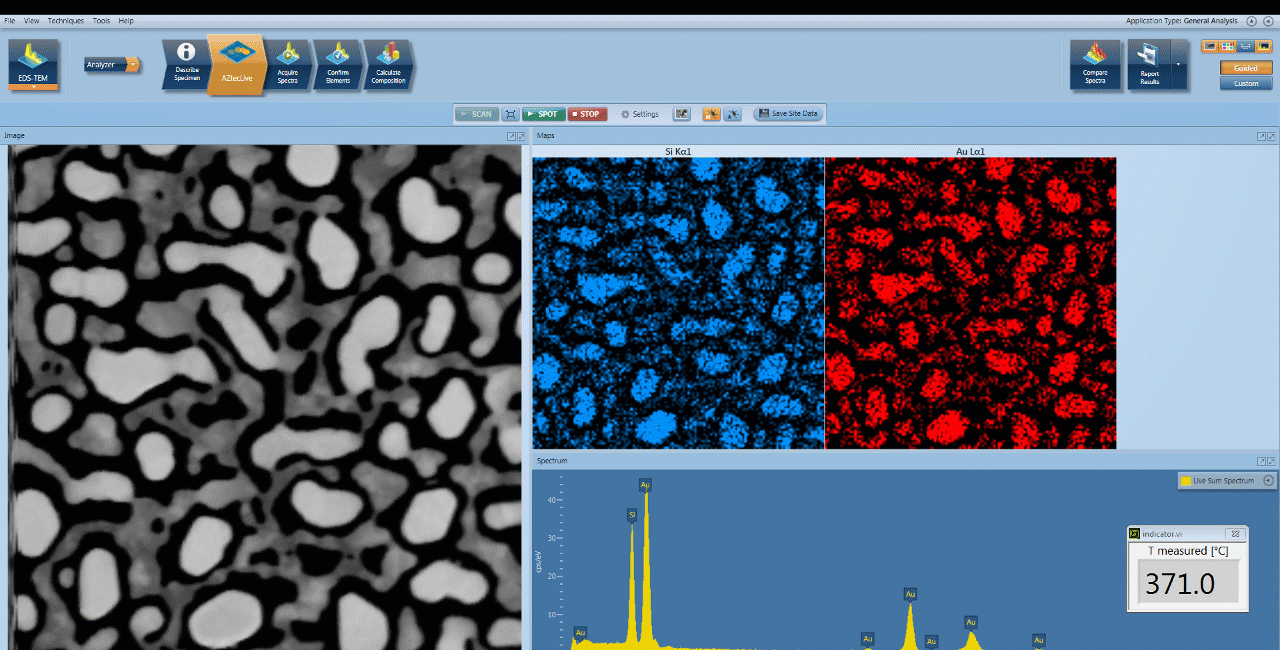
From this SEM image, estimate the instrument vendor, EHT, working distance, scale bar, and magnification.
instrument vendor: other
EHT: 80 kV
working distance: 15 mm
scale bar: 500 nm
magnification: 120 K X